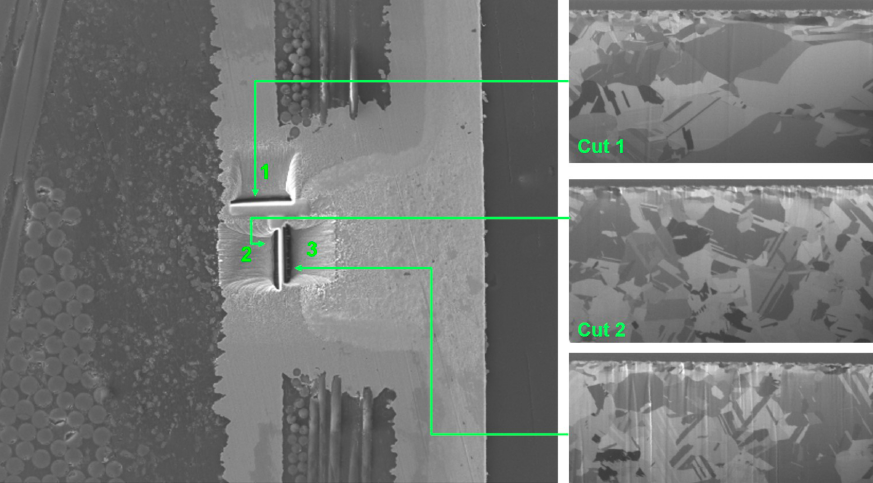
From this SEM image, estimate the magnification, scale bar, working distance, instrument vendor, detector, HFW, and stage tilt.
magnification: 1 K X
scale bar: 50000 nm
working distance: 15.6 mm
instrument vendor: FEI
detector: ETD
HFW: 298 µm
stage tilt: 0°